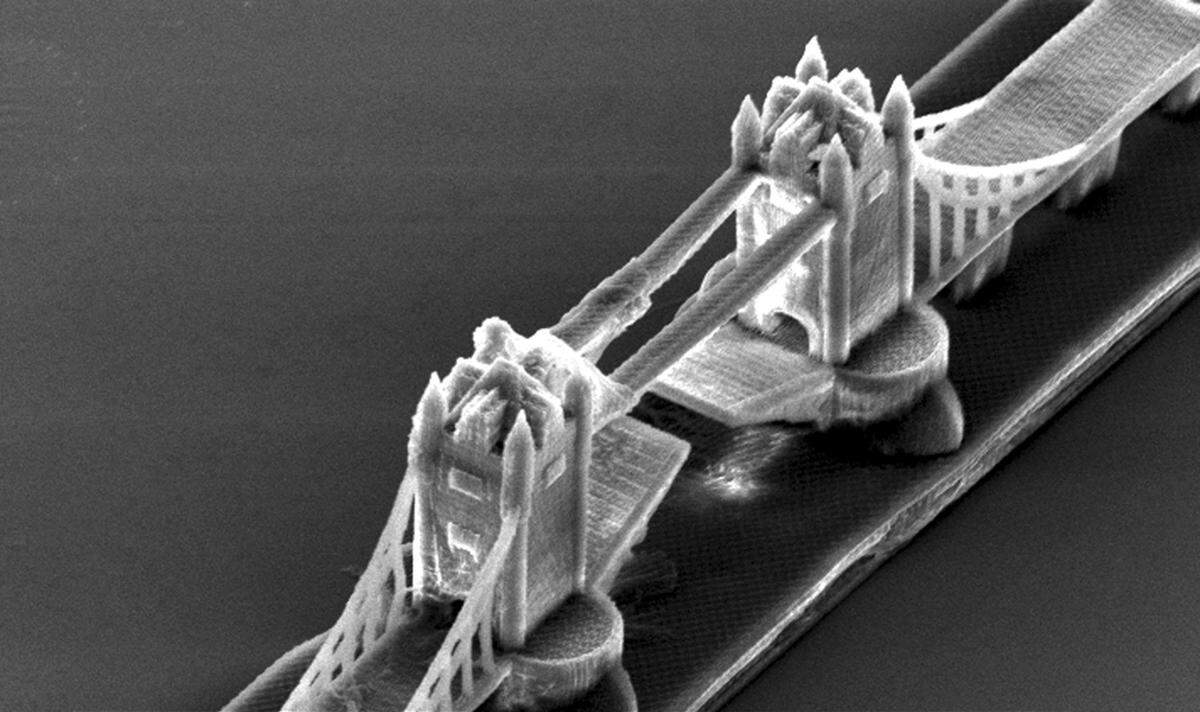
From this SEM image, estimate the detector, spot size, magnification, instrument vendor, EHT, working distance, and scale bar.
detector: SE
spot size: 4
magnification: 0.636 K X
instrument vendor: FEI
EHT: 15 kV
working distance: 27.1 mm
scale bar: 50000 nm